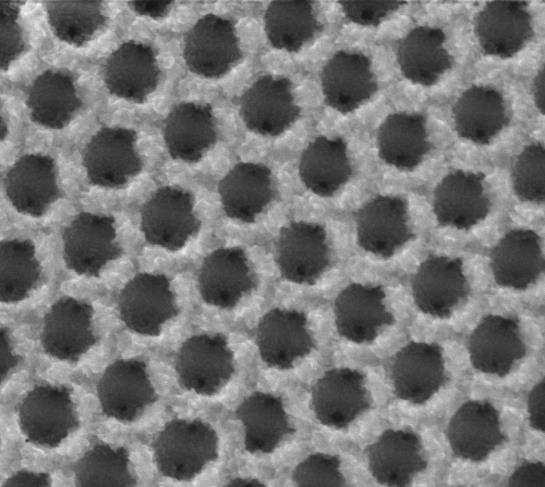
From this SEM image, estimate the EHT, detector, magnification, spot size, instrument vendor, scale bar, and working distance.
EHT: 10 kV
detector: SE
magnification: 100 K X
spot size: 2.5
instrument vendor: FEI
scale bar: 1000 nm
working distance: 9.8 mm